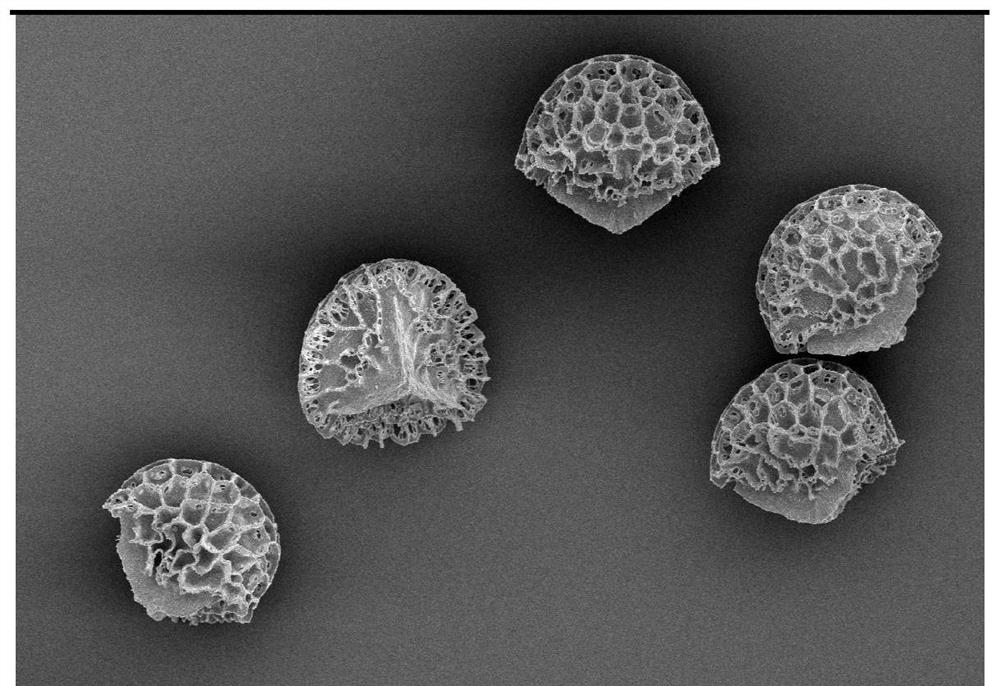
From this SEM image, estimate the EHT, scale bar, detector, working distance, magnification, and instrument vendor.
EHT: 3 kV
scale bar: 50000 nm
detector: SE(U)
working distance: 8.2 mm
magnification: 0.8 K X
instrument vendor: Hitachi